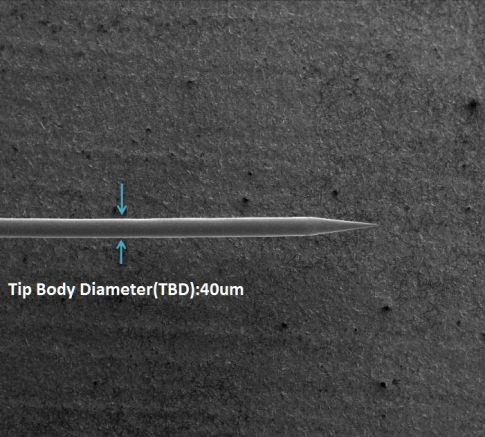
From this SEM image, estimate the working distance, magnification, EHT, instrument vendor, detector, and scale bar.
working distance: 5 mm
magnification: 0.08 K X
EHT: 5 kV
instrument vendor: FEI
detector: ETD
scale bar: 1000000 nm